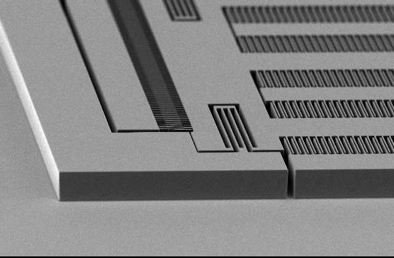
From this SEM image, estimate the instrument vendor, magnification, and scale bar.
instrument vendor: other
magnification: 0.1 K X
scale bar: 100000 nm